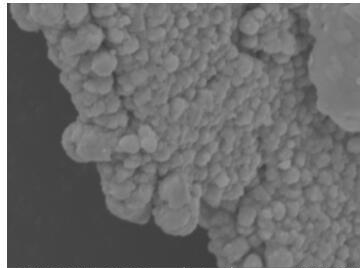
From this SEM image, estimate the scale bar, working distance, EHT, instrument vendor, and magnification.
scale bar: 2000 nm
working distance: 15.09 mm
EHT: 15 kV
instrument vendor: Tescan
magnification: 10 K X